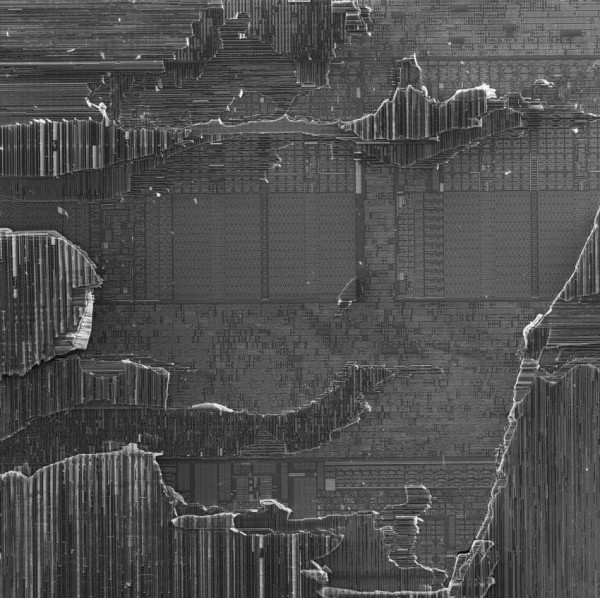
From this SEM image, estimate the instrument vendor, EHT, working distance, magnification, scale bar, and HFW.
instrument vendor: Tescan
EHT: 10 kV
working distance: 25.39 mm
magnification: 4.87 K X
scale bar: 100000 nm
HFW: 415 µm